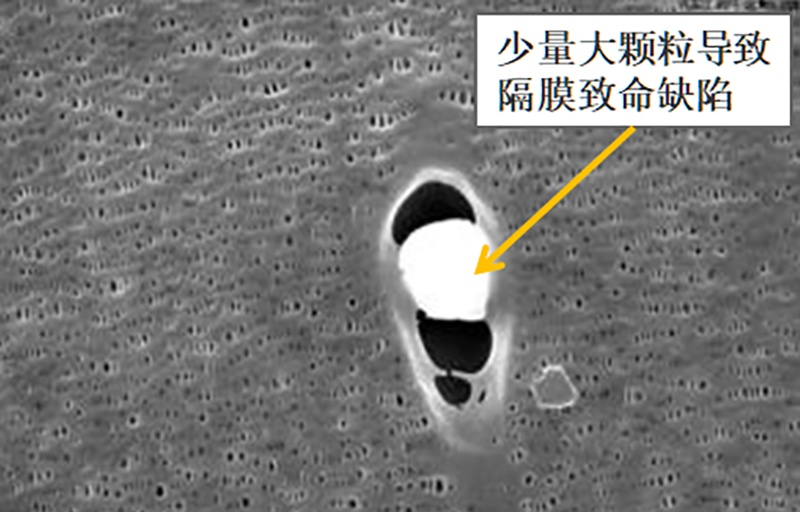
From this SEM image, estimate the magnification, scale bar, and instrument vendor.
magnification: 10 K X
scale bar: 1000 nm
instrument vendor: JEOL